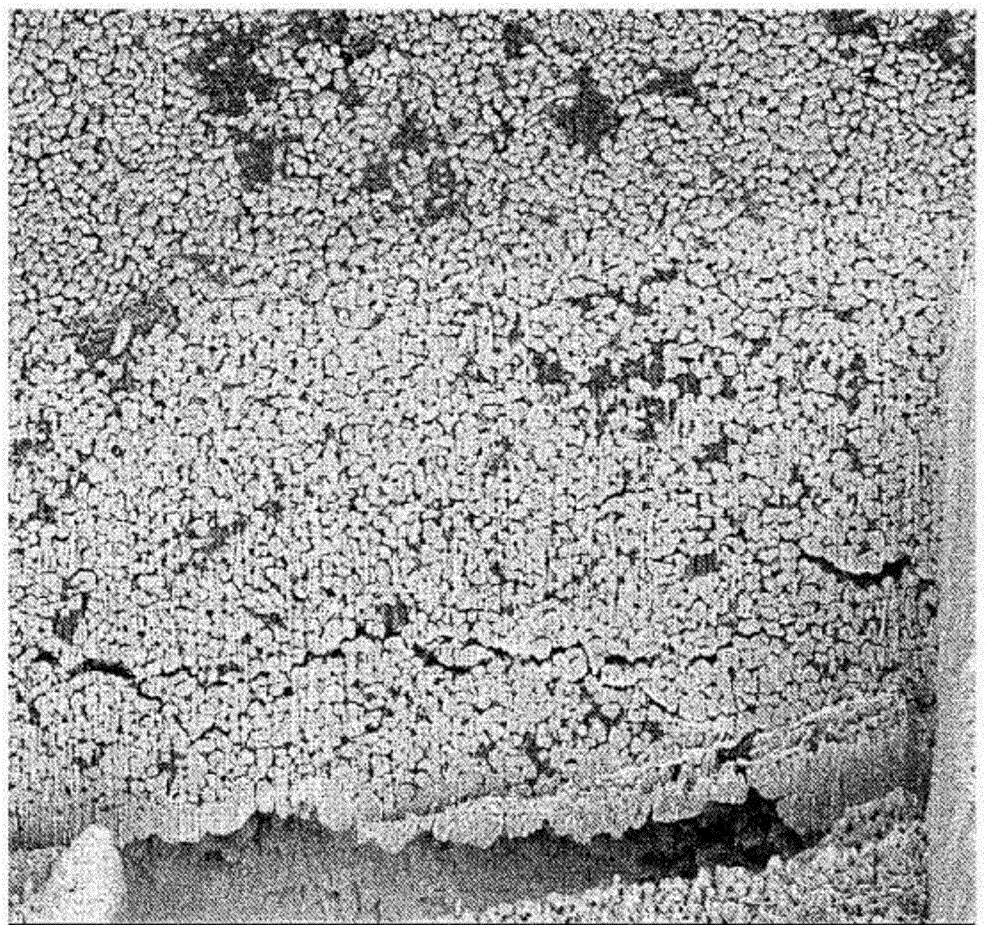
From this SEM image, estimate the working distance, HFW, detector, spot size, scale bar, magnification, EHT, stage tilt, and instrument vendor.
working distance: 4.996 mm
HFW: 60.8 µm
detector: TLD-B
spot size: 3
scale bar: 10000 nm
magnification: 2.5 K X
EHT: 5 kV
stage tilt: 52°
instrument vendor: FEI